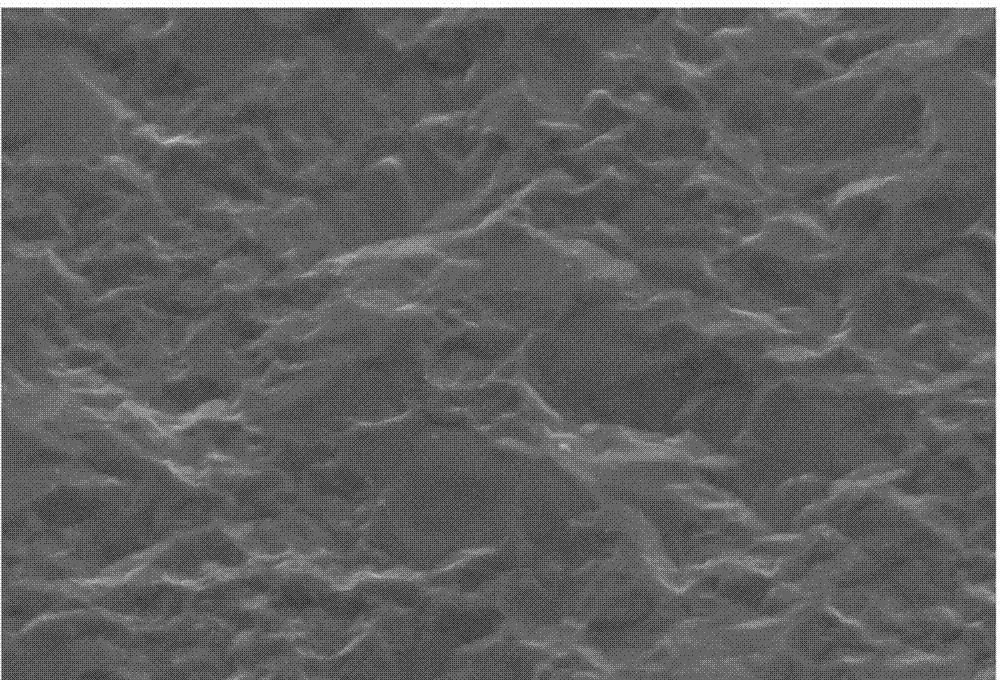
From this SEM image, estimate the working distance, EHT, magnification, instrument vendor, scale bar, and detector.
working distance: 19 mm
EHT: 15 kV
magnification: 3 K X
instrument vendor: Hitachi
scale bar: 10000 nm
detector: SE